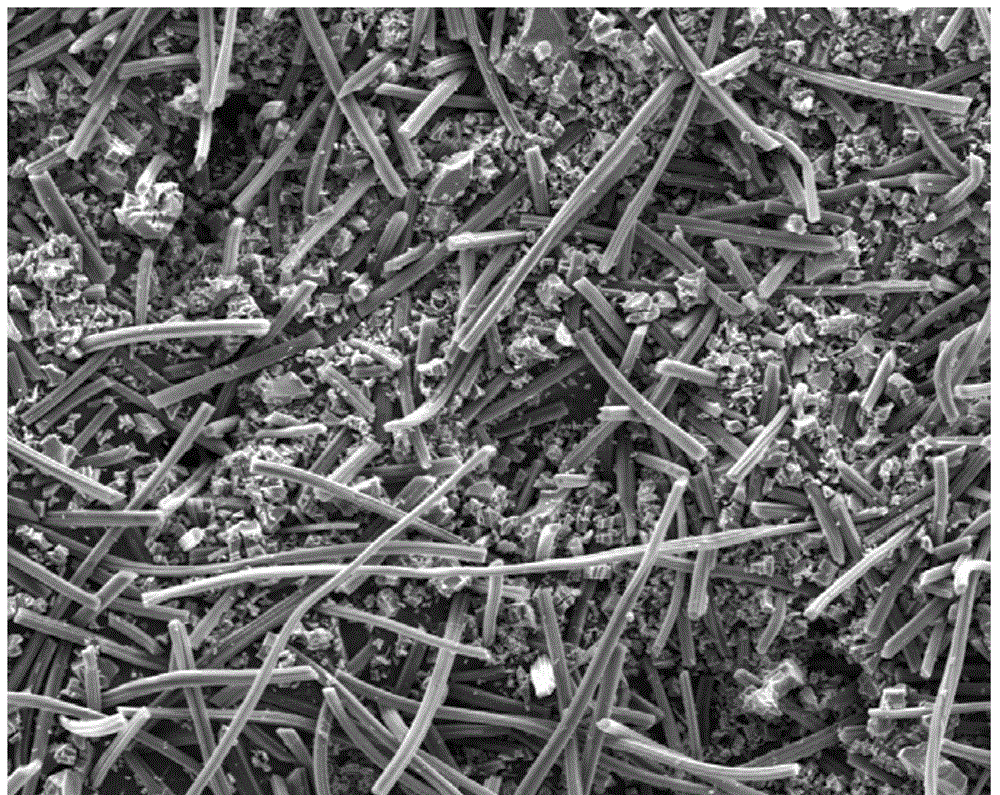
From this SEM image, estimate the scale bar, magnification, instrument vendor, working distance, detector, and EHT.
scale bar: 300000 nm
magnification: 0.4 K X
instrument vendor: FEI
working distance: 11.6 mm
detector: ETD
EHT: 20 kV